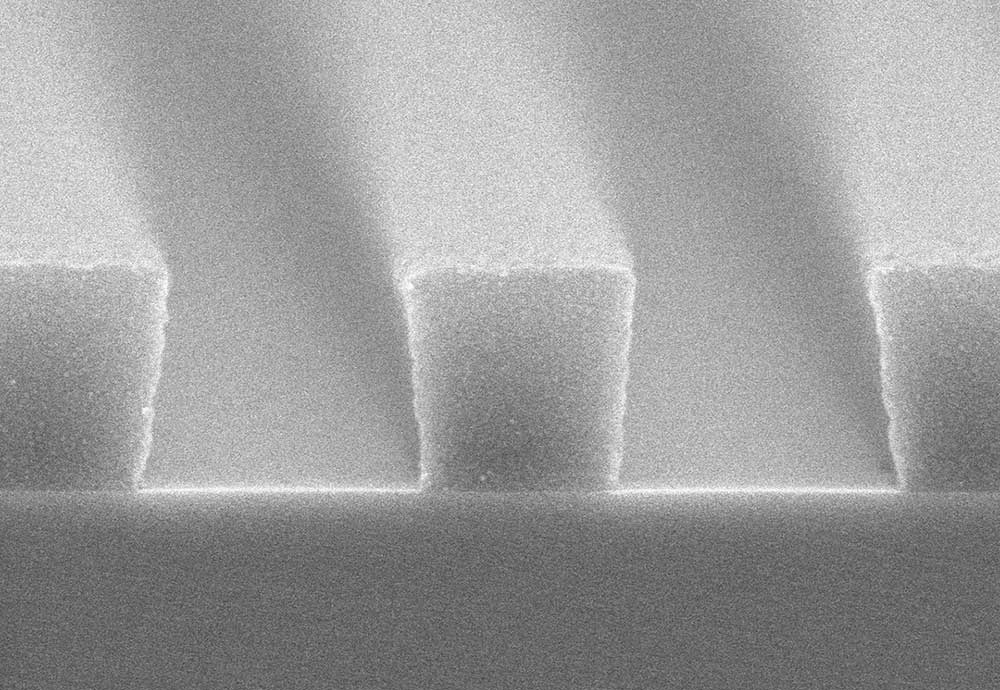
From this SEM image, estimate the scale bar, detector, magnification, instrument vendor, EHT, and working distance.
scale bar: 500 nm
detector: SE(UL)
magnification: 60 K X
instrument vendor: Hitachi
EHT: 5 kV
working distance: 8 mm